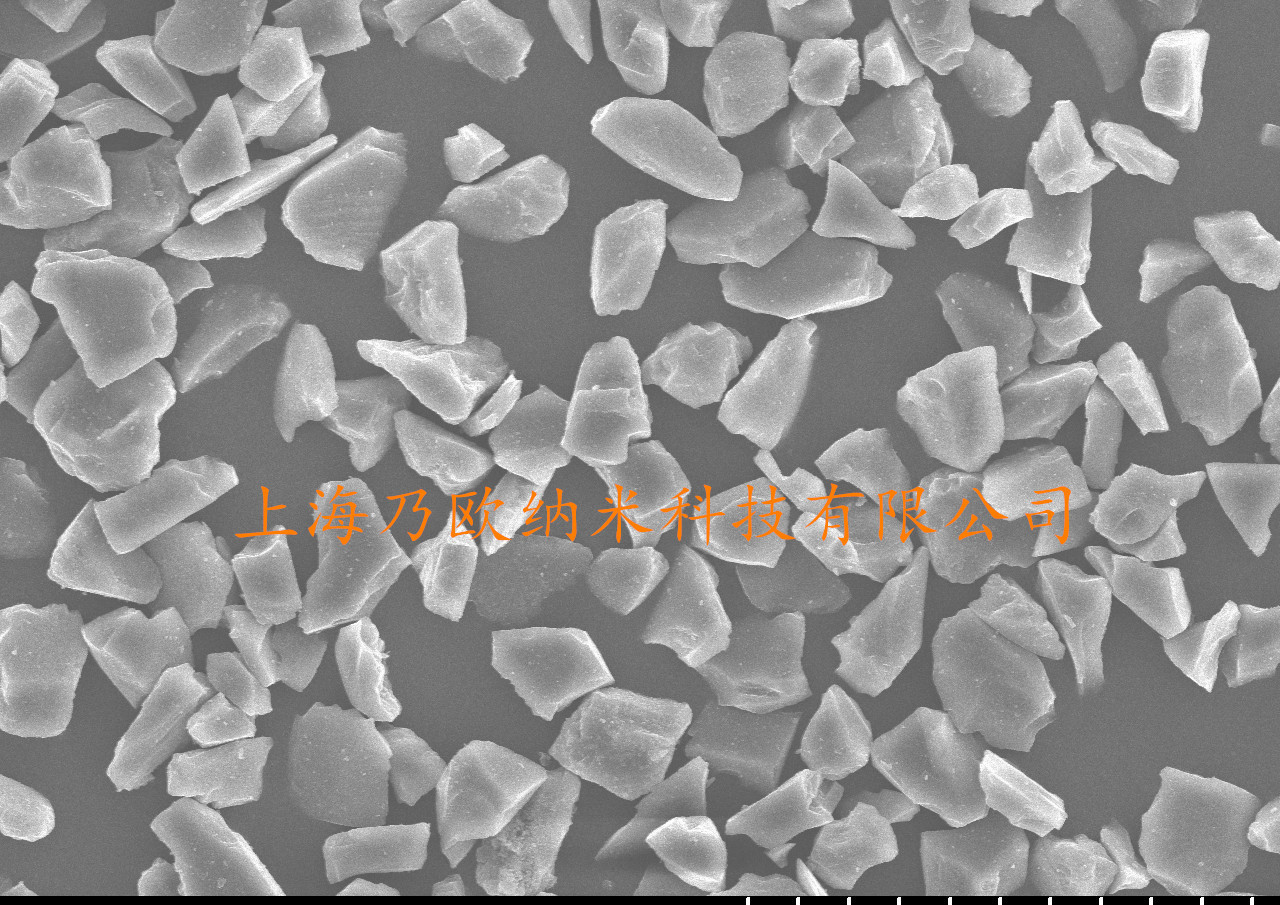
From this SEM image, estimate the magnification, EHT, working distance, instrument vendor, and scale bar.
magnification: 1 K X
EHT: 20 kV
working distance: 13.2 mm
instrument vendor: Hitachi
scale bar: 50000 nm